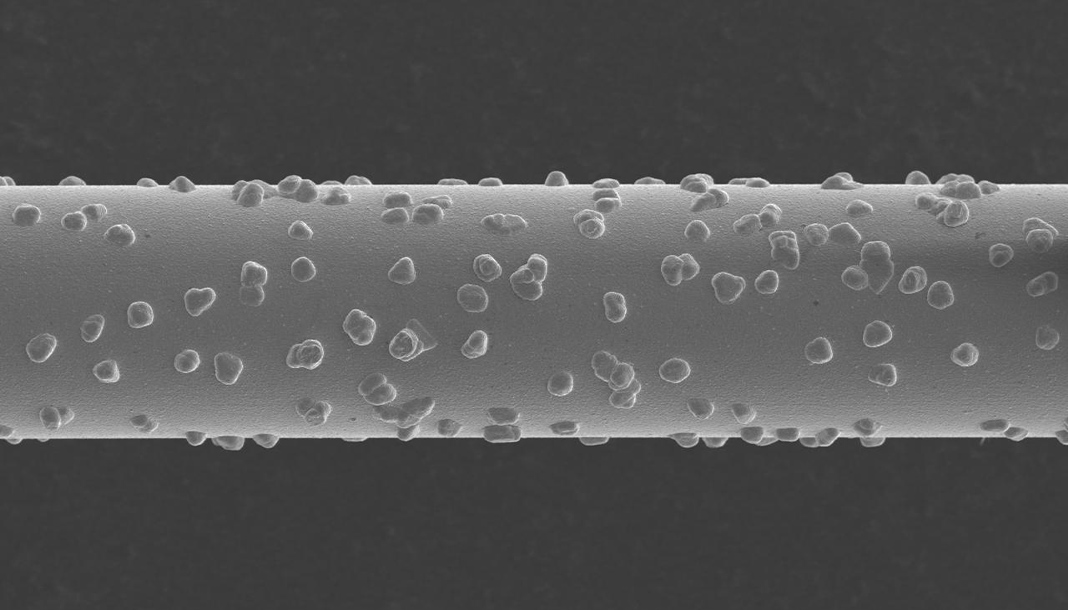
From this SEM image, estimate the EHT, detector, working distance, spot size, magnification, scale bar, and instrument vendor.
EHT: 10 kV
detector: SEI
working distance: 10 mm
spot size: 50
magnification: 0.25 K X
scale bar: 100000 nm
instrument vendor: JEOL